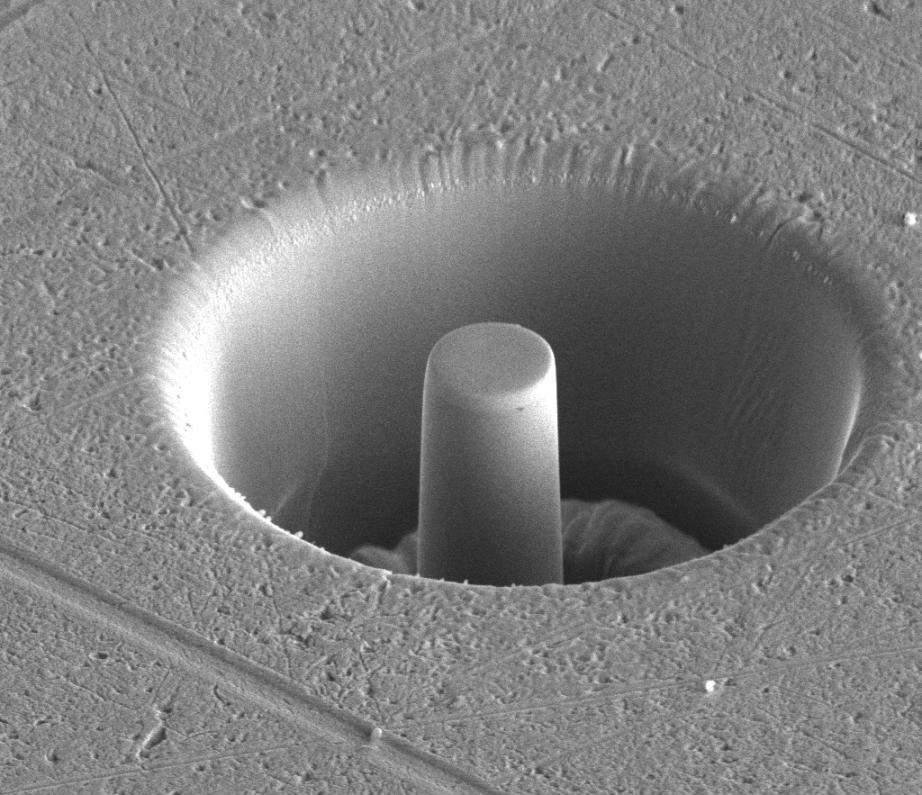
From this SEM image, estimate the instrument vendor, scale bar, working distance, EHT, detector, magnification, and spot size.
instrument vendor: FEI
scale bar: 5000 nm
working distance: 10 mm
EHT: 5 kV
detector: ETD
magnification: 7.502 K X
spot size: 4